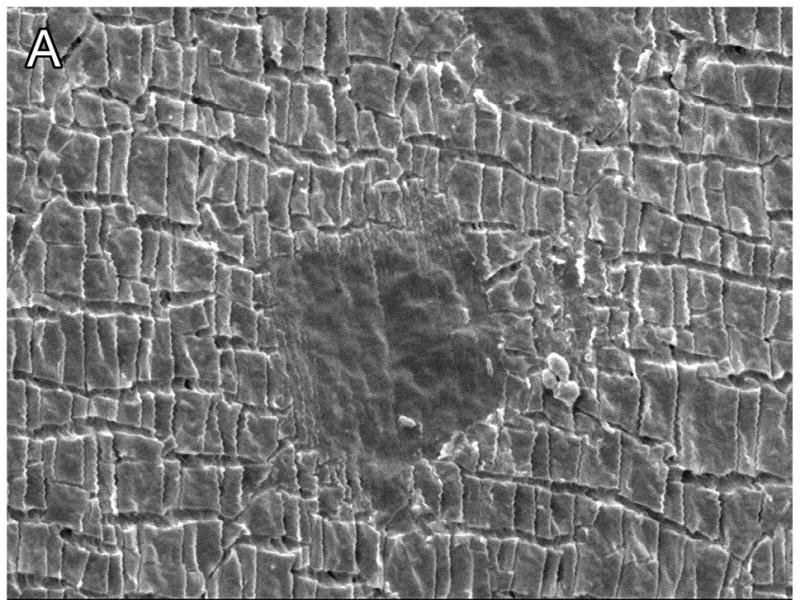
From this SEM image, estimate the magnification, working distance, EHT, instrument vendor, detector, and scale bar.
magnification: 2 K X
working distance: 7.7 mm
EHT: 5 kV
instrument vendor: JEOL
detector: LEI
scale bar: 10000 nm